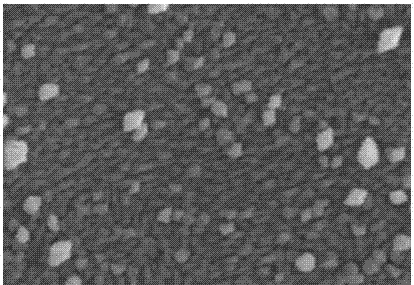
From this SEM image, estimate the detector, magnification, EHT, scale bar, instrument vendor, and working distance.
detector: SE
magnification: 20 K X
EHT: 15 kV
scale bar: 2000 nm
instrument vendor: Hitachi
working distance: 11.6 mm